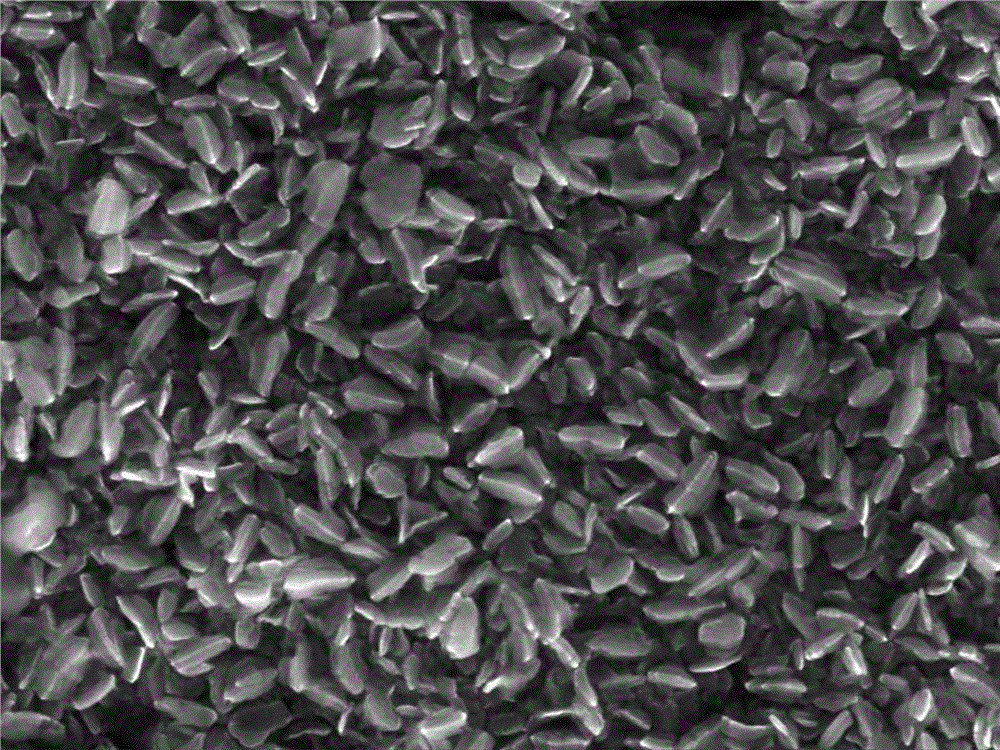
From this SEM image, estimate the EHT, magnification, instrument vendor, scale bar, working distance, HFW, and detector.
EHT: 10 kV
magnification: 50 K X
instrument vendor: Tescan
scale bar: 1000 nm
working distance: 5.94 mm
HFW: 4.33 µm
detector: InBeam